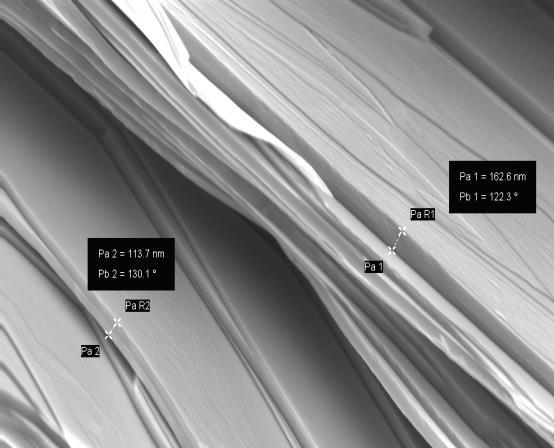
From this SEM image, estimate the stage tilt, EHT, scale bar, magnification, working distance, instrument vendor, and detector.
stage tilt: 0°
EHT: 5 kV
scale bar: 200 nm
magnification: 24.38 K X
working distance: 4.7 mm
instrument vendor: Zeiss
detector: InLens